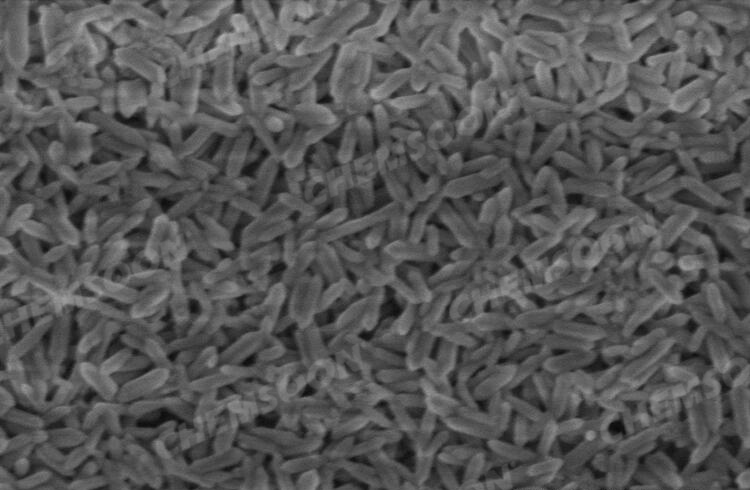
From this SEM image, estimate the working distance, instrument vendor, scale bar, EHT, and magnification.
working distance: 3.6 mm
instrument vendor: Hitachi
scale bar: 500 nm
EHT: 1 kV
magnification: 60 K X